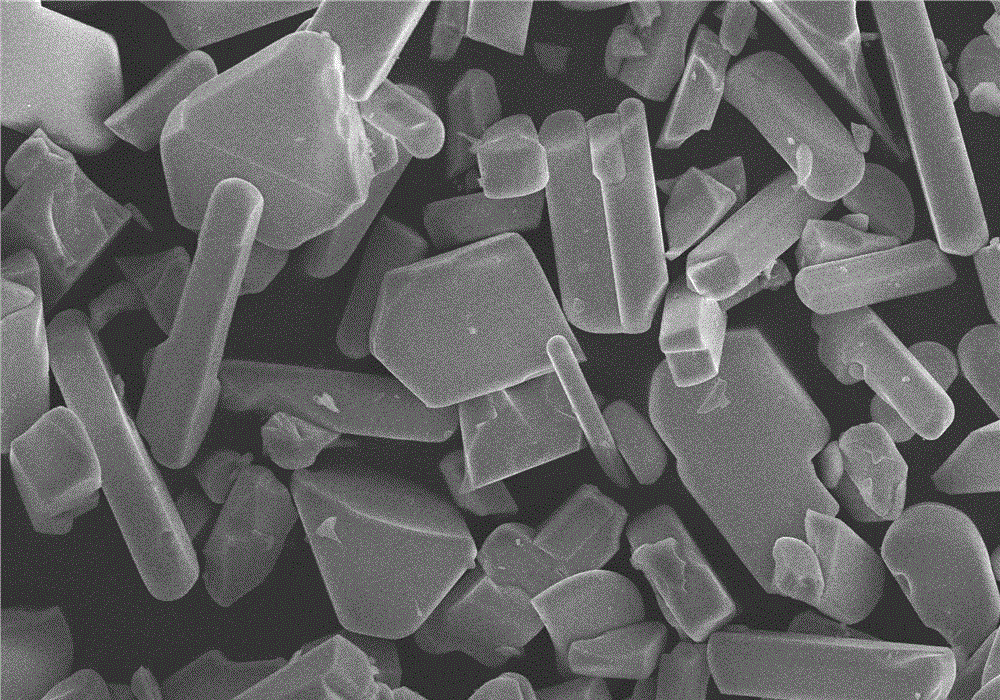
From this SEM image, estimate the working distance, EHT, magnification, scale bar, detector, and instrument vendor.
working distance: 8.1 mm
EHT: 10 kV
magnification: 2 K X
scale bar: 20000 nm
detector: SE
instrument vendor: Hitachi